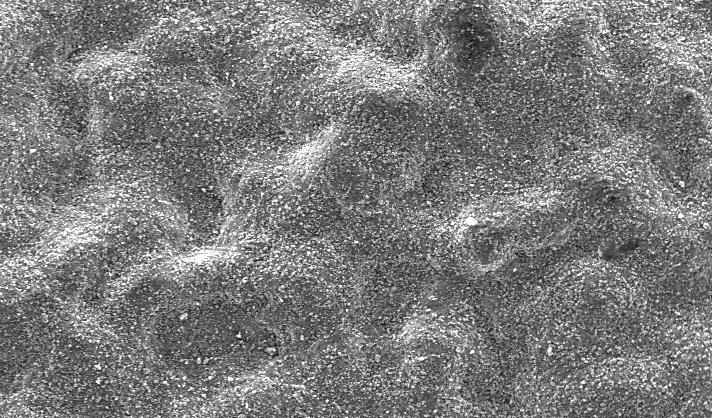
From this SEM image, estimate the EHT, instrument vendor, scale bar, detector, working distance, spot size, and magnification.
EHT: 20 kV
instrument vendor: FEI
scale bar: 100000 nm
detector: SE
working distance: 10.2 mm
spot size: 4.2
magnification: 0.65 K X